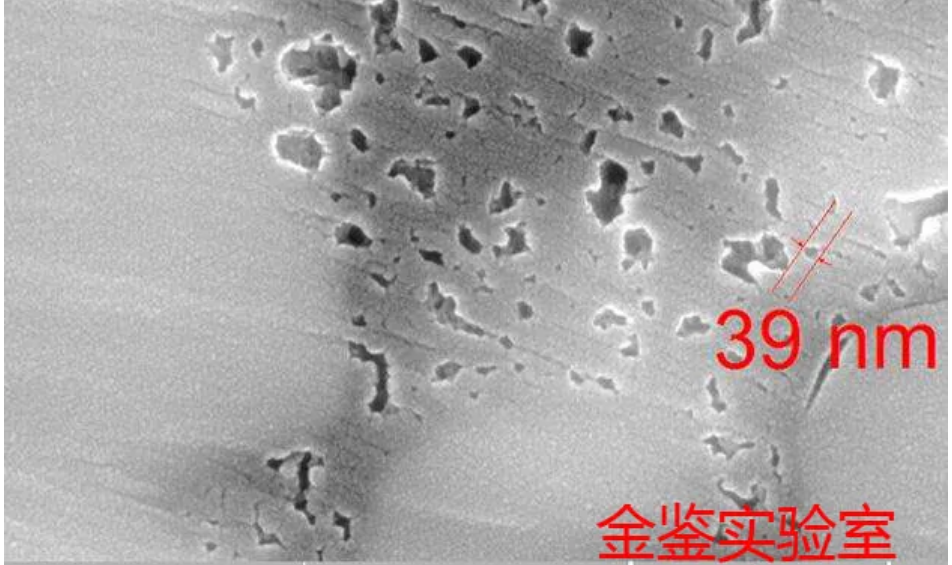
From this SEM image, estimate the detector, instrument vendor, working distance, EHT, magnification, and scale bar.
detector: InBeam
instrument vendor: Tescan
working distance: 6.03 mm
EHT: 20 kV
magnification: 100 K X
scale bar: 500 nm